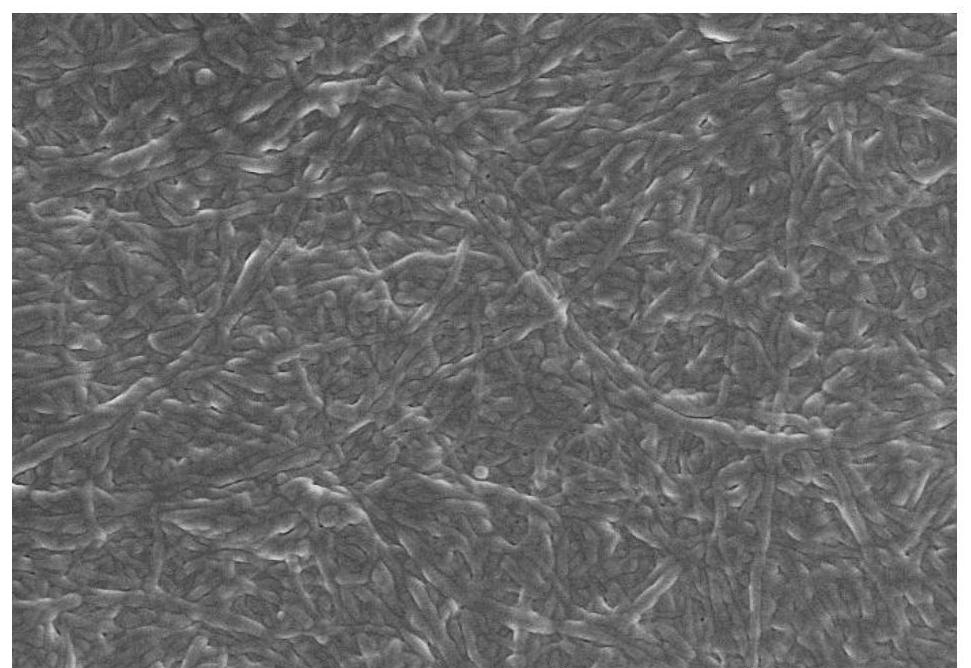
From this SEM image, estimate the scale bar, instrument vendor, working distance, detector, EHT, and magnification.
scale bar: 5000 nm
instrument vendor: Hitachi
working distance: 8.5 mm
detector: SE(TUL)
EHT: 3 kV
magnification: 10 K X